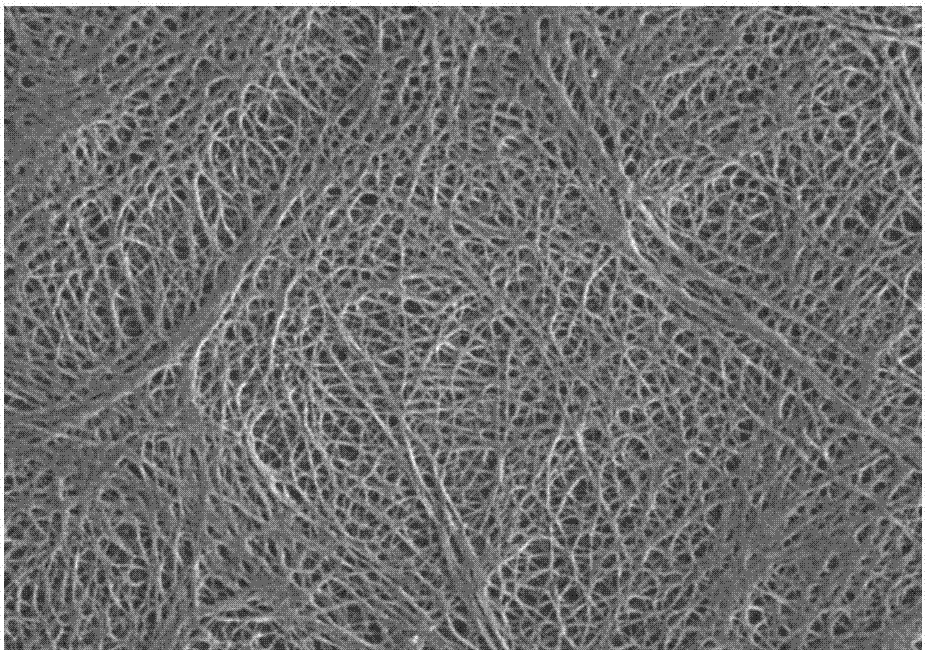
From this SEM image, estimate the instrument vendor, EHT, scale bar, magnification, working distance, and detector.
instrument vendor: Zeiss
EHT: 1 kV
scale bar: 200 nm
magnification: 10 K X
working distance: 3.4 mm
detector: SE2